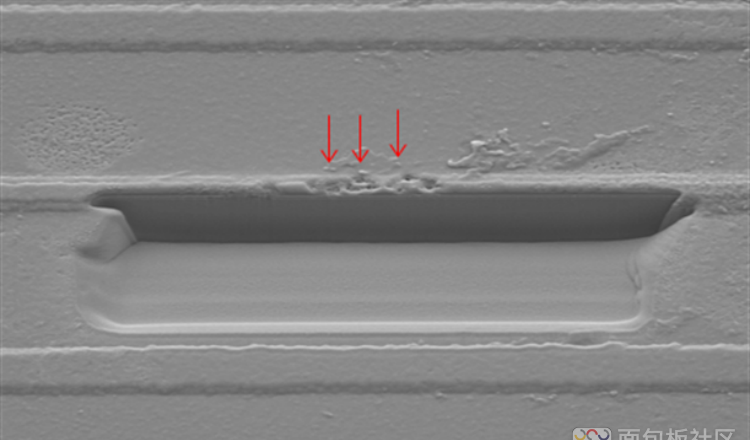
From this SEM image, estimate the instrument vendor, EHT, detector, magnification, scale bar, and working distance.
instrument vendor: Hitachi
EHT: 5 kV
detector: SE(M)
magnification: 3 K X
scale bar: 10000 nm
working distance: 18.1 mm